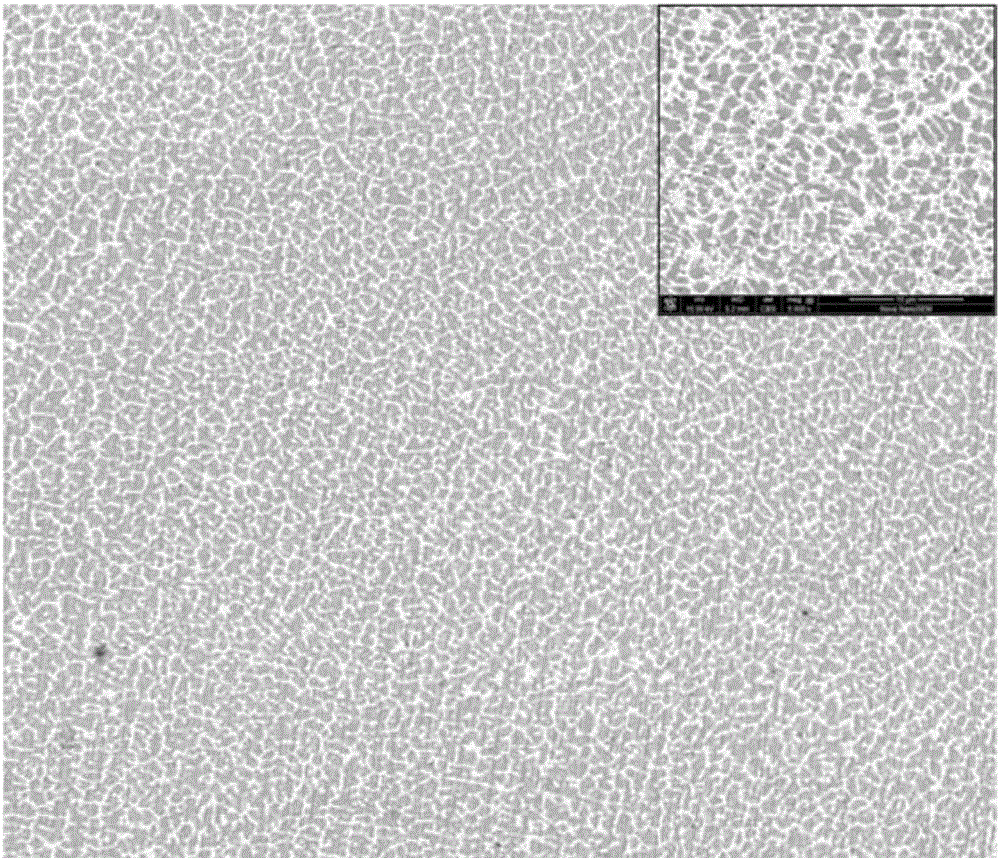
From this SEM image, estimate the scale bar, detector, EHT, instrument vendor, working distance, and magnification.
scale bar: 50000 nm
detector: CBS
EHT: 10 kV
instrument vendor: FEI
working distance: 5.7 mm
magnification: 0.8 K X